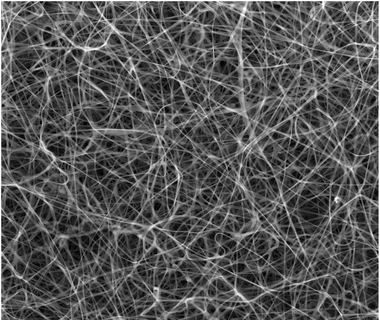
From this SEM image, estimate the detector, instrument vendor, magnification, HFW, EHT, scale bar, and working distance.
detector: LFD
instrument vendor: FEI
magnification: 0.8 K X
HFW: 373 µm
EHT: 20 kV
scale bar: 100000 nm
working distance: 8 mm